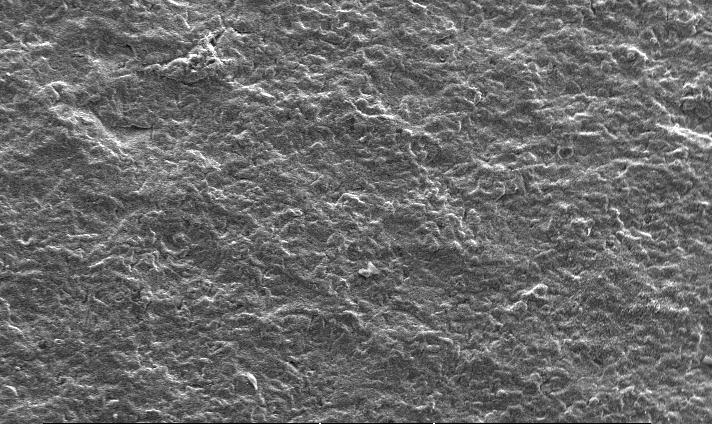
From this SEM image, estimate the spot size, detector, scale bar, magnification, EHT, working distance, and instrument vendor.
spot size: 3.5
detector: SE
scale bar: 200000 nm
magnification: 0.1 K X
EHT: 25 kV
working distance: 6.6 mm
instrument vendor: FEI